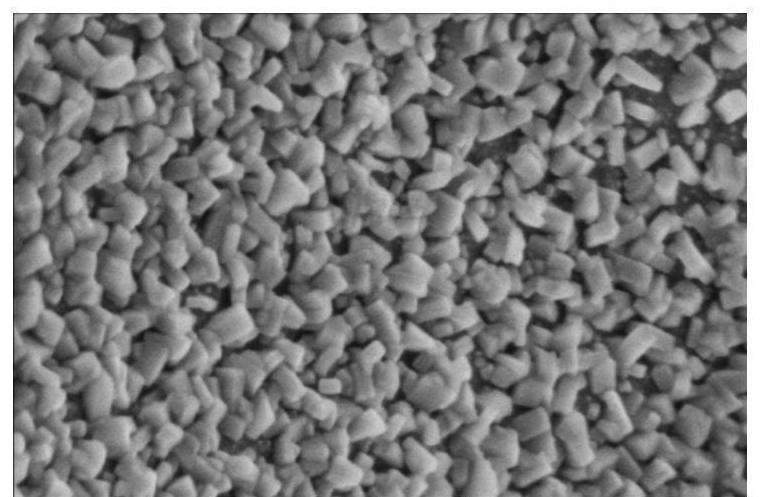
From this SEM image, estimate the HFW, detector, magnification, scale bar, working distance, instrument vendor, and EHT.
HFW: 1.38 µm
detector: TLD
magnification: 150 K X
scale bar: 300 nm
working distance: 3.5 mm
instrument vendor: FEI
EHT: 2 kV